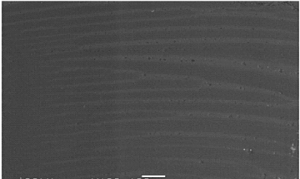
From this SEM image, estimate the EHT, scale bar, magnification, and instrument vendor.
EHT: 20 kV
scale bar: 100000 nm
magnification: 0.1 K X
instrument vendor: JEOL